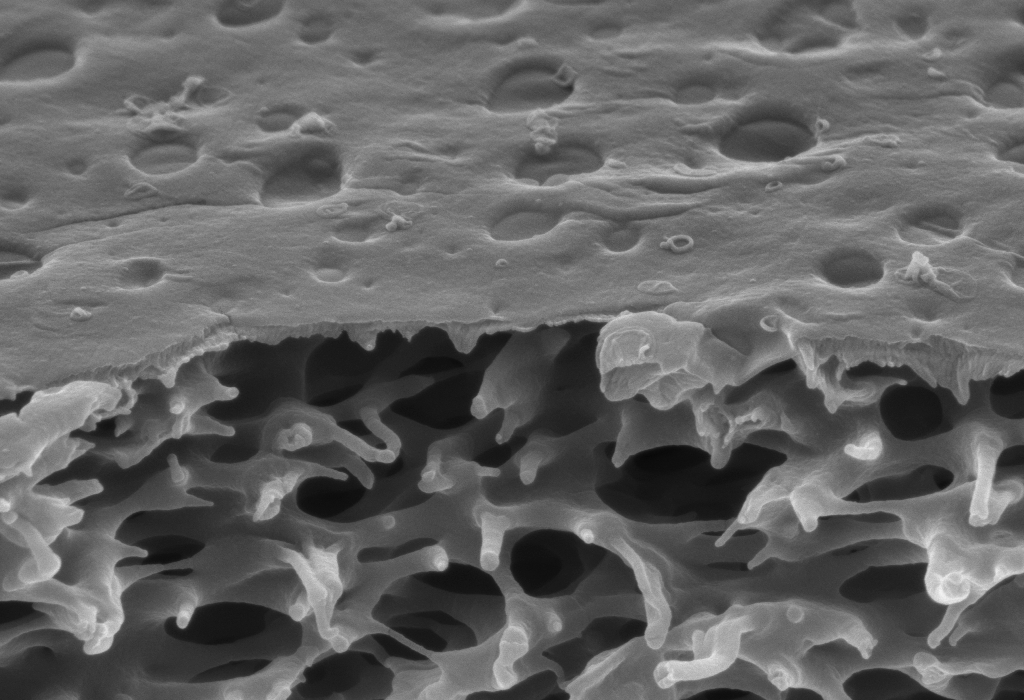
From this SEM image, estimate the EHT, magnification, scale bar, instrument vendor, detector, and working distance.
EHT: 5 kV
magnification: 50 K X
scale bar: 1000 nm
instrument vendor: Zeiss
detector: InLens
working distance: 4.2 mm